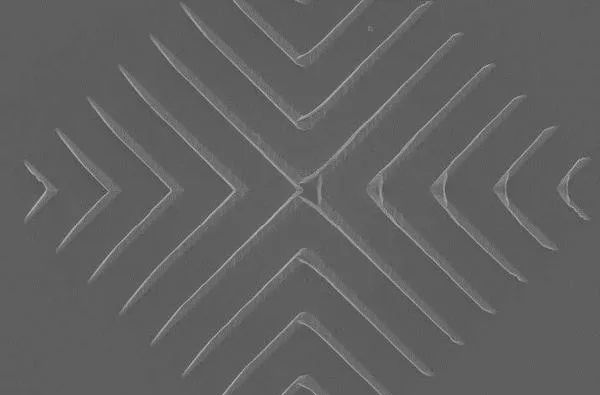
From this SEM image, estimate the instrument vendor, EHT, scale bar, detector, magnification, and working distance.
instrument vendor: Zeiss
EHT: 3 kV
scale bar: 2000 nm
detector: InLens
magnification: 10.51 K X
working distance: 3.3 mm